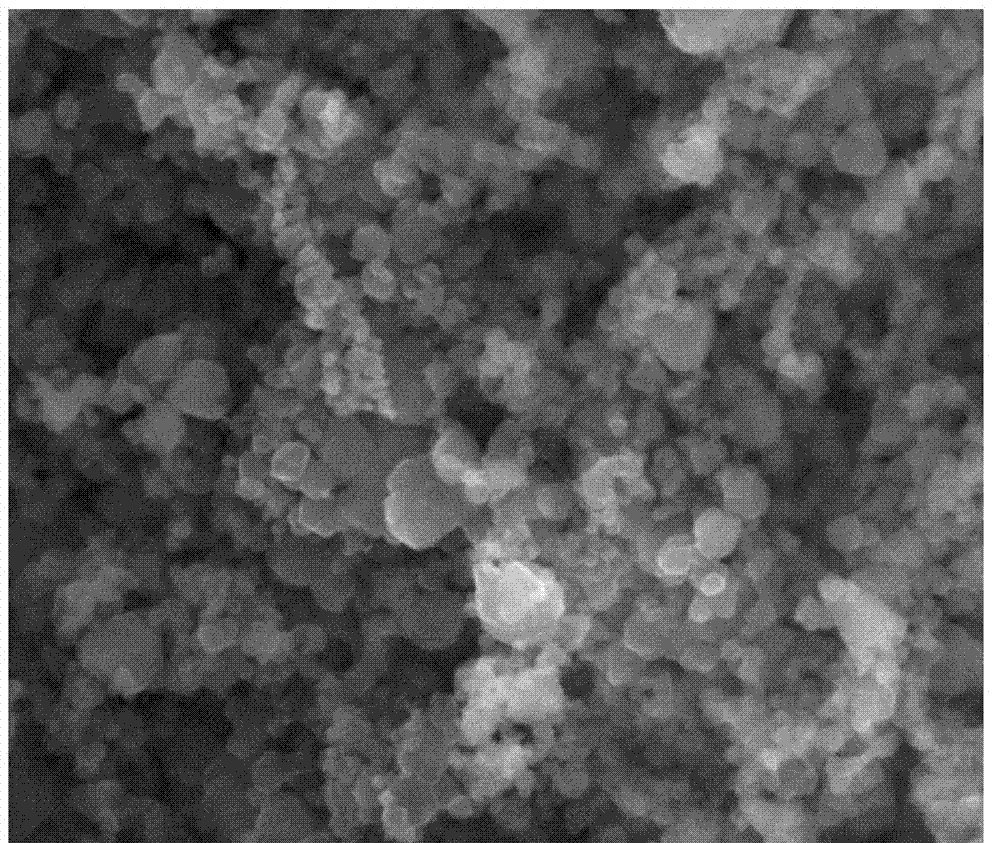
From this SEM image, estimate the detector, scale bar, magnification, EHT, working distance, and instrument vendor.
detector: SE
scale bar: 500 nm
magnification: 50 K X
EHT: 20 kV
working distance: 4.3 mm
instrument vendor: FEI